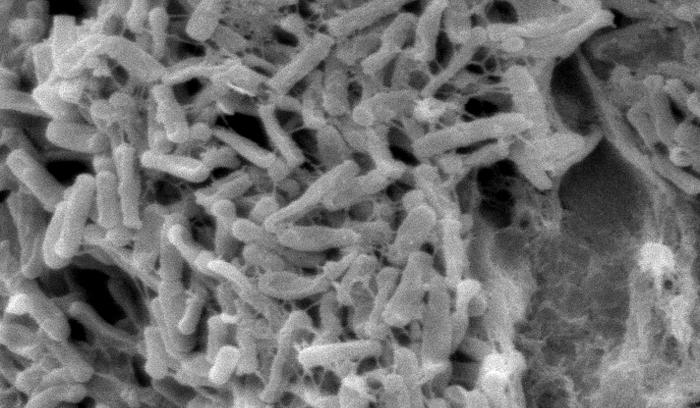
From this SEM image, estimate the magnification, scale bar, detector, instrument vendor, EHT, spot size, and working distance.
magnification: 4.595 K X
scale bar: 5000 nm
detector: SE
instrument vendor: FEI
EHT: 10 kV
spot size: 3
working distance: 33.6 mm